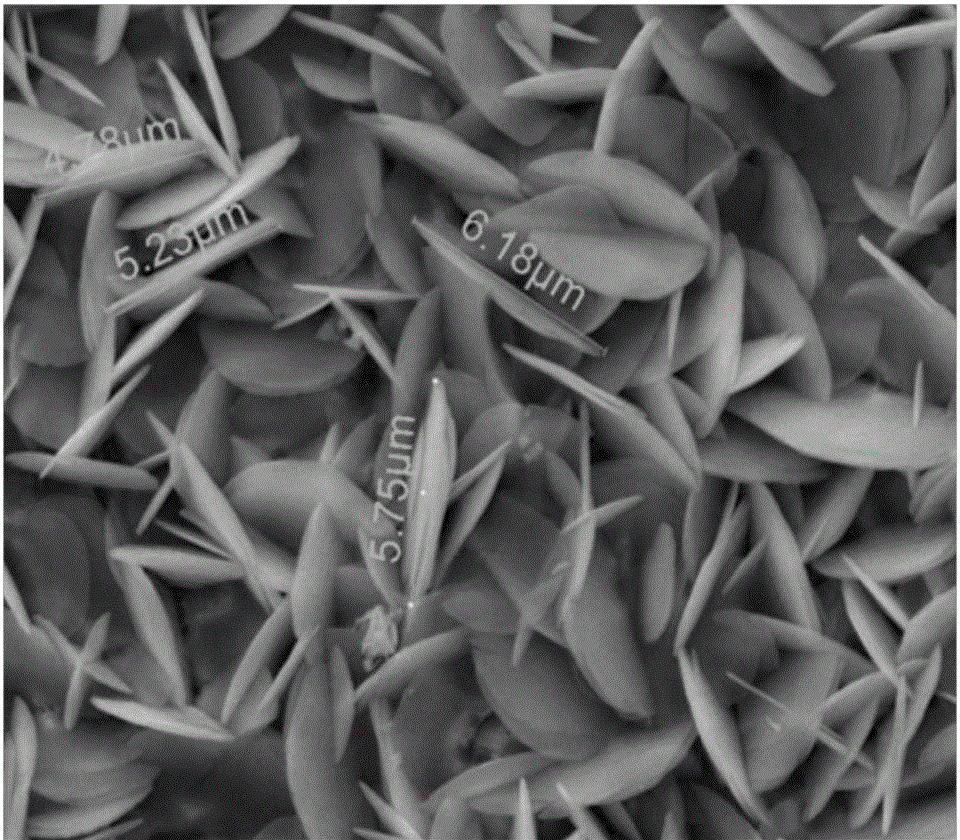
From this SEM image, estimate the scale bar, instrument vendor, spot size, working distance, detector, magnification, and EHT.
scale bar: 5000 nm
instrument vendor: FEI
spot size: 4.5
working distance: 10.7 mm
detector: ETD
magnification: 10 K X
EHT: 15 kV